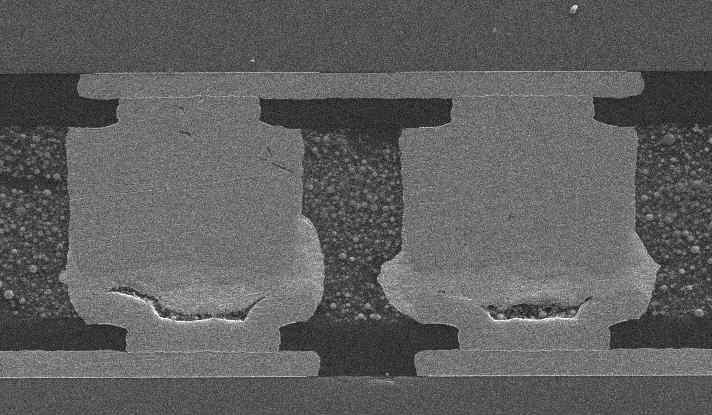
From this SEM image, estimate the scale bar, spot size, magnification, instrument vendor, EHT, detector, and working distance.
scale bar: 20000 nm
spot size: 3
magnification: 1 K X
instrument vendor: FEI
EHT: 10 kV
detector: SE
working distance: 12.5 mm